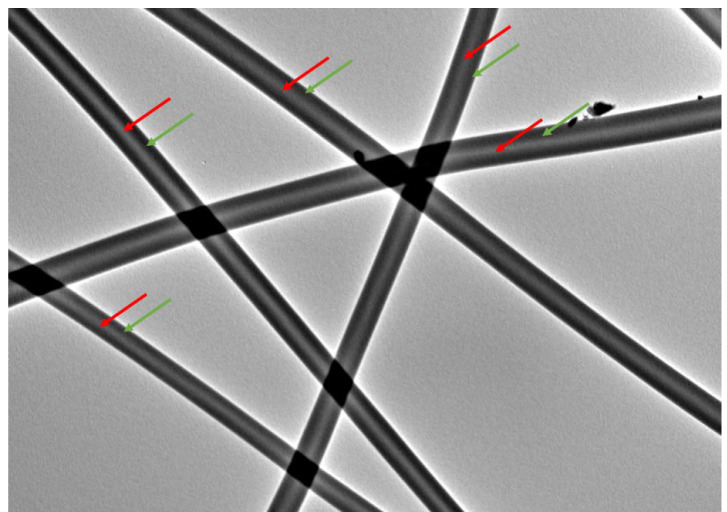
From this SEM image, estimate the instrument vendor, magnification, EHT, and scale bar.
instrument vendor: JEOL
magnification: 5 K X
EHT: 120 kV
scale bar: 5000 nm